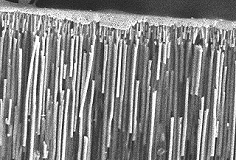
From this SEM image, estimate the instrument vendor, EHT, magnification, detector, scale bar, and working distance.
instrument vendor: Hitachi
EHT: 10 kV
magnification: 6.08 K X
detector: SE(M)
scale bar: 5000 nm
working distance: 11.4 mm